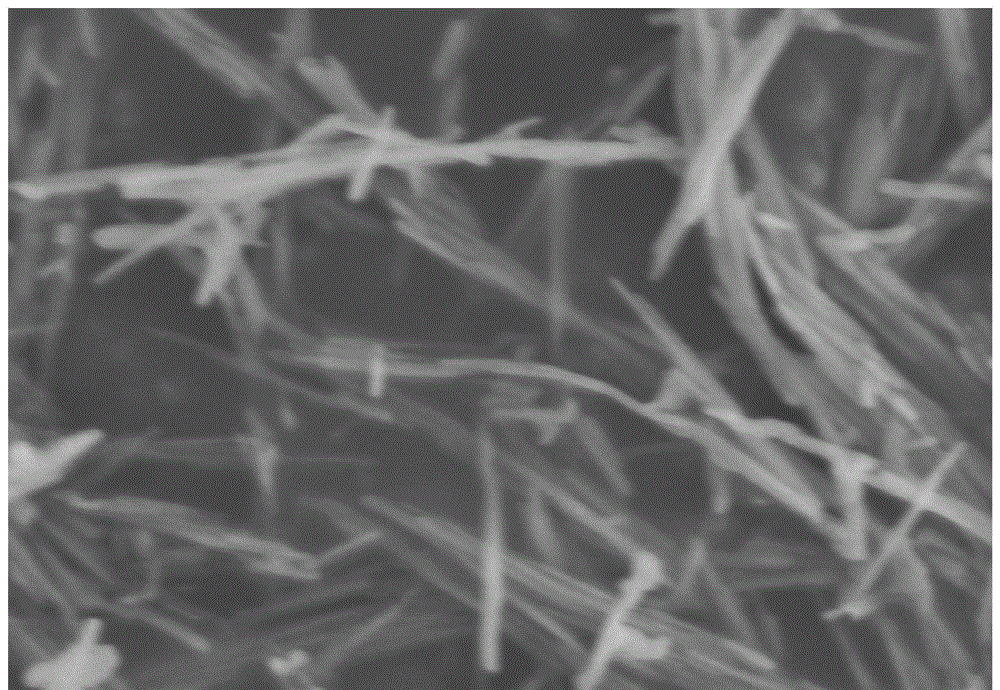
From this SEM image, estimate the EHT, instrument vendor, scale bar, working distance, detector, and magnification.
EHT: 5 kV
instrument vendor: Hitachi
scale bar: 1000 nm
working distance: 15.6 mm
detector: SE(M)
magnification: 30 K X